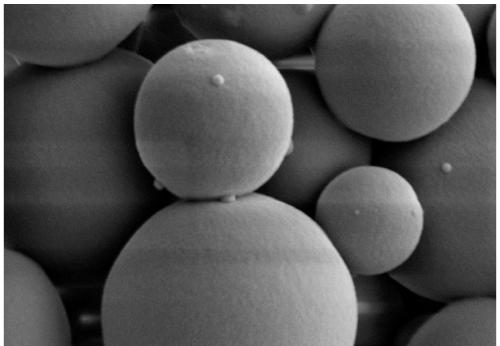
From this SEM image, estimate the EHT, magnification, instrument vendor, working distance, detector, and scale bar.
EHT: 4 kV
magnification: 20 K X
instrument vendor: Zeiss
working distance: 9.5 mm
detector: SE2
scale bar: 200 nm